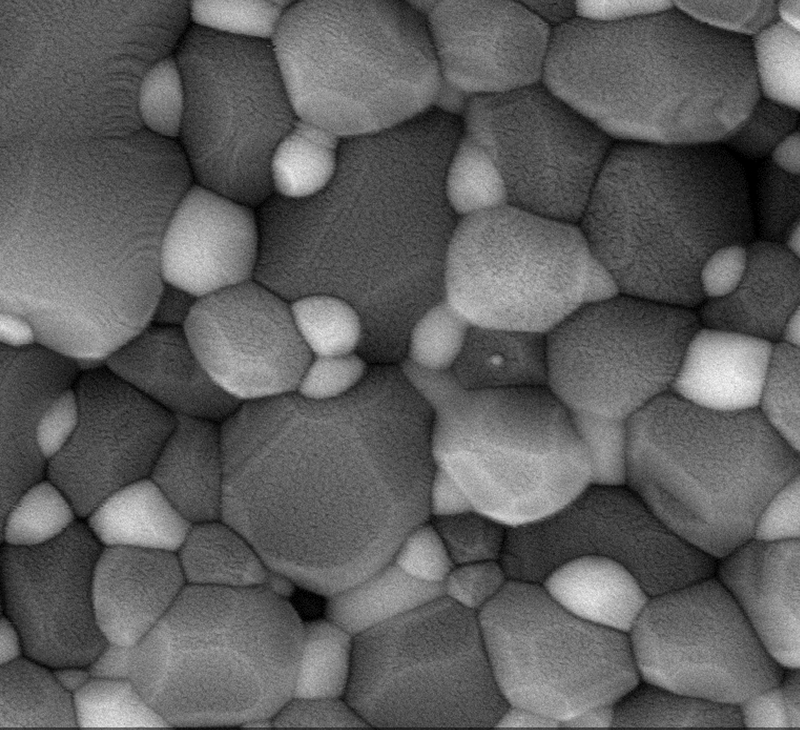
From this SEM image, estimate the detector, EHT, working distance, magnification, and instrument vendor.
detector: SE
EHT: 10 kV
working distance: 9.5 mm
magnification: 50 K X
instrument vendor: other